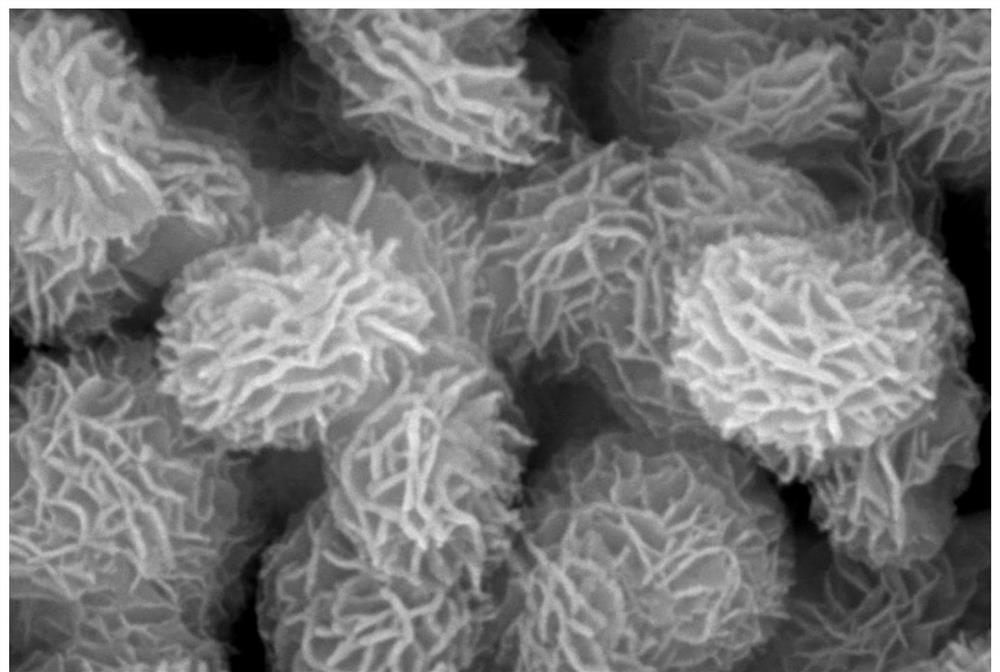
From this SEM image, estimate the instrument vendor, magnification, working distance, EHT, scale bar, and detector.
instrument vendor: Zeiss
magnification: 50 K X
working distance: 8.3 mm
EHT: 20 kV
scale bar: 100 nm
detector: InLens